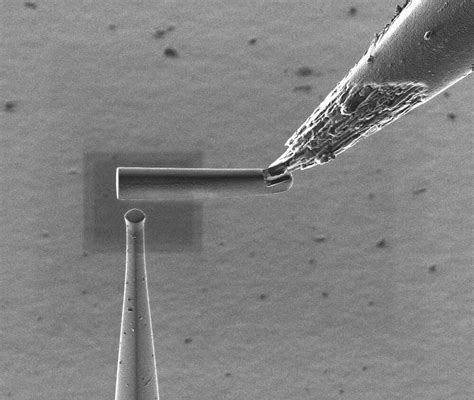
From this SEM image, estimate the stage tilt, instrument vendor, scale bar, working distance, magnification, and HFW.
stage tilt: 0°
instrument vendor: FEI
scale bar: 20000 nm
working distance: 16.3 mm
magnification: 5 K X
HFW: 51.2 µm